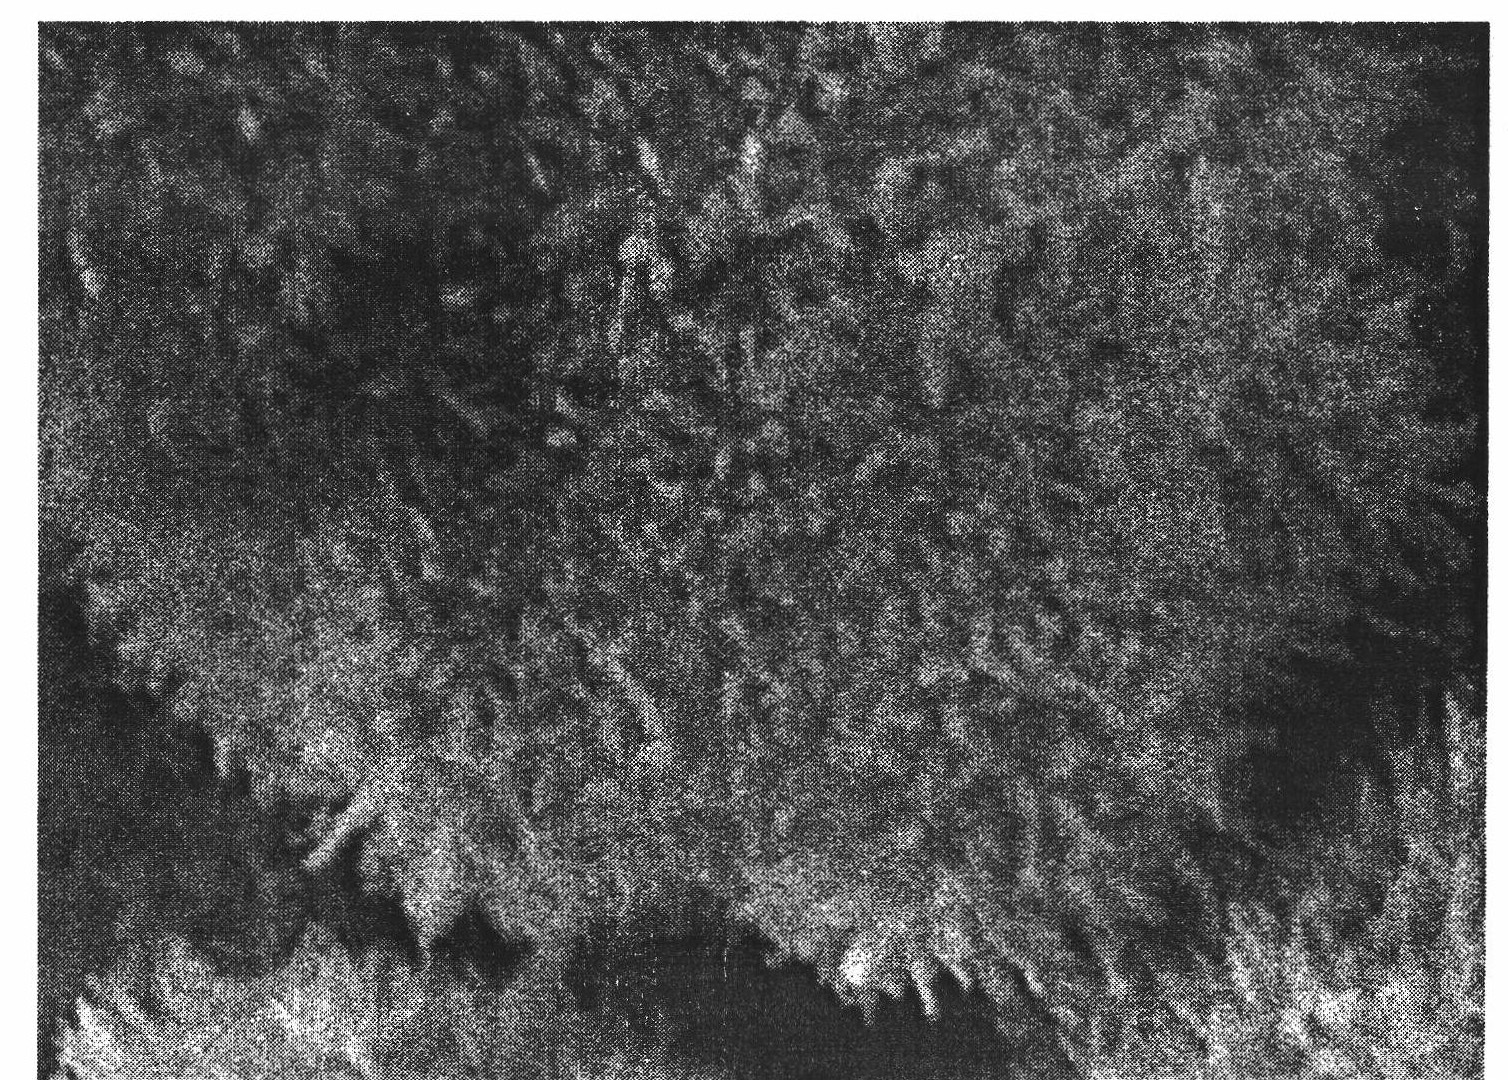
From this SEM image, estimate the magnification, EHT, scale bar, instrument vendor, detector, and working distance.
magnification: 40 K X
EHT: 5 kV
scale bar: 100 nm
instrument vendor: JEOL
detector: SEI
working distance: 6 mm